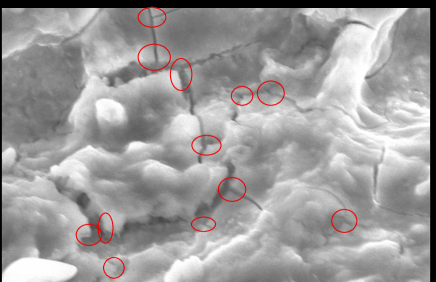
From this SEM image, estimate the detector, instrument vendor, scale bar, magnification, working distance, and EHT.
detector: SE(U)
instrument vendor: Hitachi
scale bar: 5000 nm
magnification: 10.1 K X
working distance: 14.7 mm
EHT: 10 kV